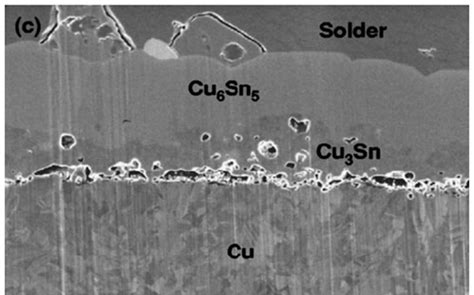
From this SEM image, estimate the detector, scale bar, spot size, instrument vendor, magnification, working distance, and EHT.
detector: TLD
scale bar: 5000 nm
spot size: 3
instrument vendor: FEI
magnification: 15 K X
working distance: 5.7 mm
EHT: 5 kV